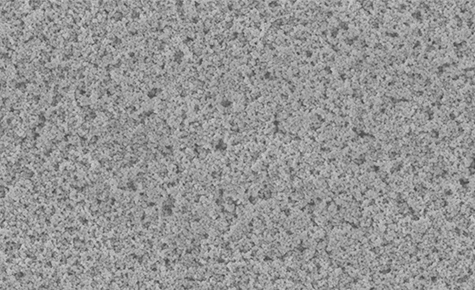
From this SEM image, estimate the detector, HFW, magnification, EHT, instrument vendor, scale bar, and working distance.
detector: SED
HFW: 35.6 µm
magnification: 14.5 K X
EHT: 10 kV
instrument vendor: Thermo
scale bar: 10000 nm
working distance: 7.697 mm